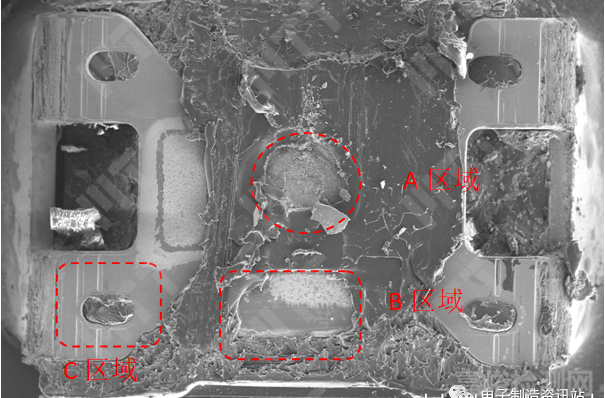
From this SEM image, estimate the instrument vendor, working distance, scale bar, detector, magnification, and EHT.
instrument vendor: Hitachi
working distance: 9.6 mm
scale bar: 1000000 nm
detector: SE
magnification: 0.035 K X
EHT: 15 kV